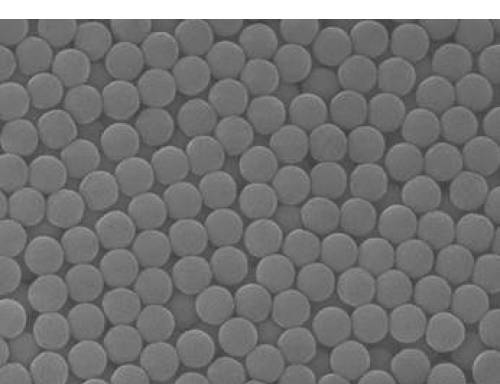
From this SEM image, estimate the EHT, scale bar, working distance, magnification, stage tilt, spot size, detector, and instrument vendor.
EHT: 20 kV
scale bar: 2000 nm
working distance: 8.8 mm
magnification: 50 K X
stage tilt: -0°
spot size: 3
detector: ETD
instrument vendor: FEI